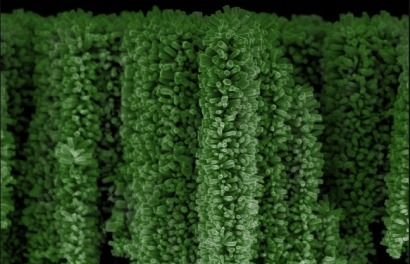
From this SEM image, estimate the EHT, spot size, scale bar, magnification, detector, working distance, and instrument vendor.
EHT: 5 kV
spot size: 2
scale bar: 1000 nm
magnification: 20 K X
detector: TLD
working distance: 4.3 mm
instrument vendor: FEI